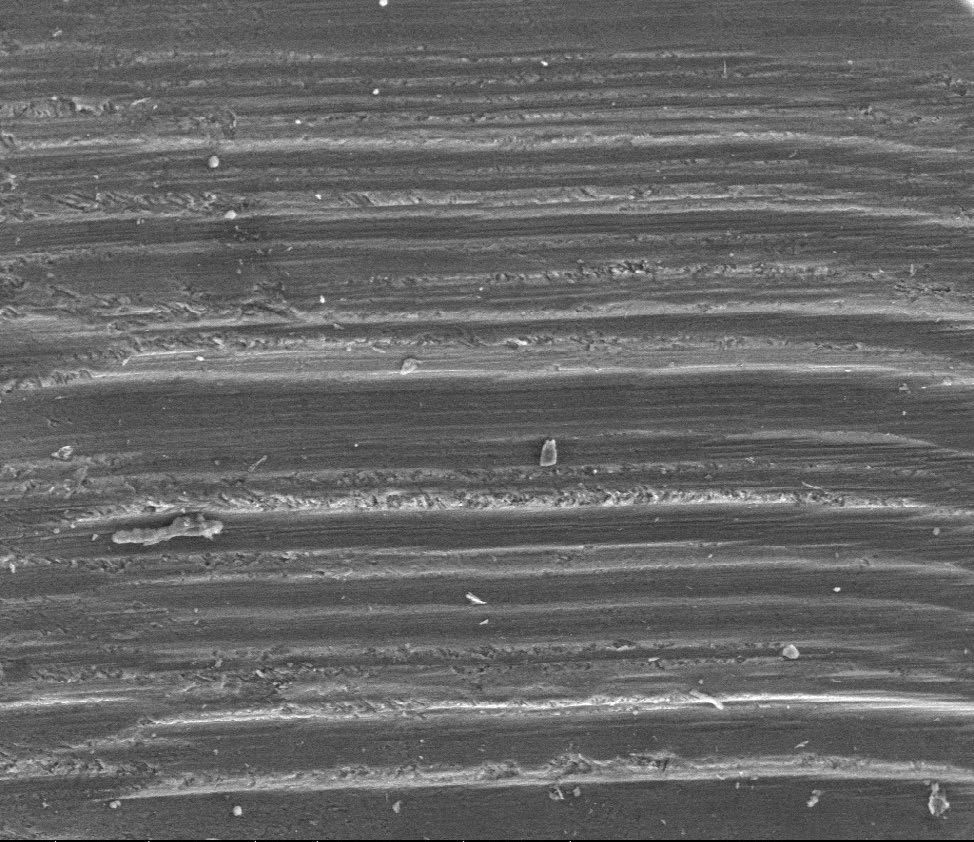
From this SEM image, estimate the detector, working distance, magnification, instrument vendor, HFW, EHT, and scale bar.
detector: LFD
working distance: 12.6 mm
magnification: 0.1 K X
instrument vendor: FEI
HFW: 1280 µm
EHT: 30 kV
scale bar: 500000 nm